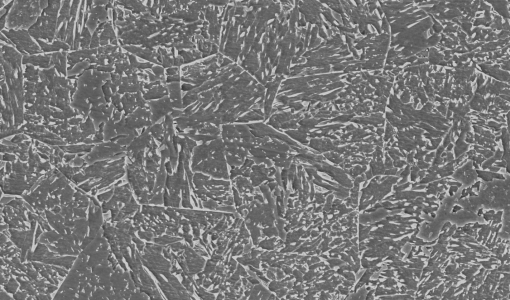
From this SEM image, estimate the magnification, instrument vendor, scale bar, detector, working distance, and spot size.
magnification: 1 K X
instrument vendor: FEI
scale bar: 20000 nm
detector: SE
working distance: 11 mm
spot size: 5